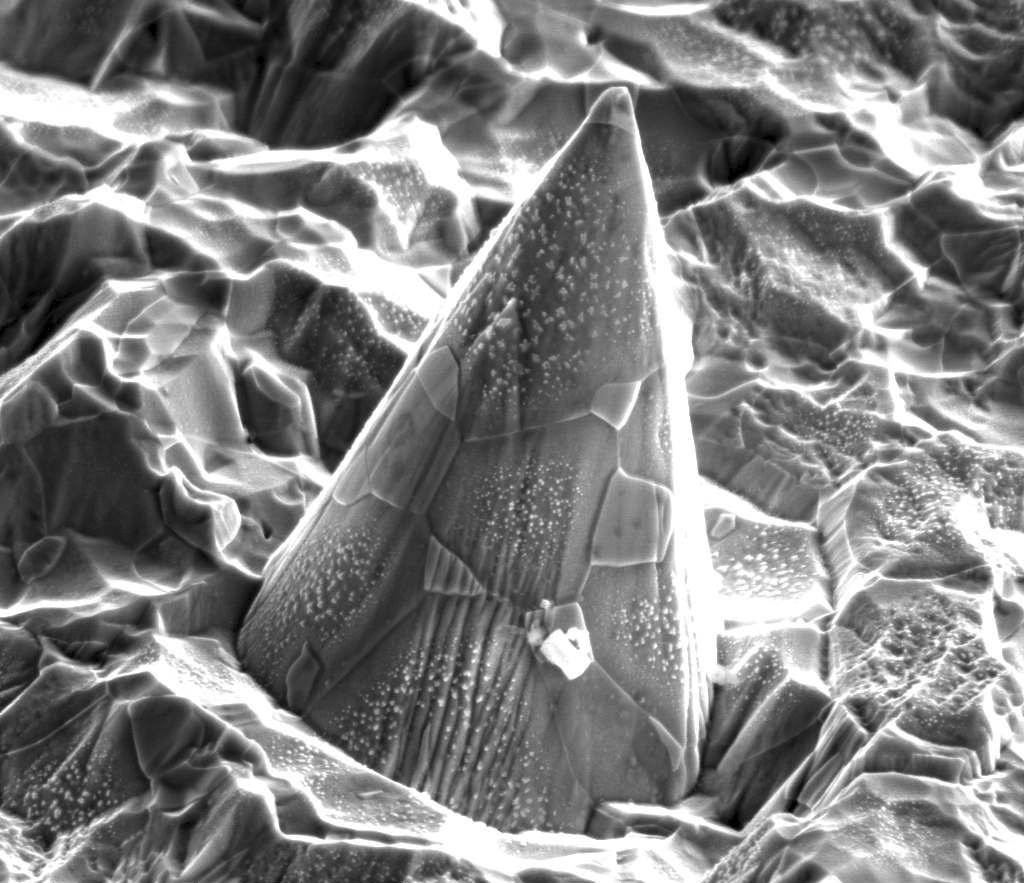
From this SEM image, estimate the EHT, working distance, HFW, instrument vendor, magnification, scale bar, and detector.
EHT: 10 kV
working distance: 10.1 mm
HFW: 24.9 µm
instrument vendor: FEI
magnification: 6 K X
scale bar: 5000 nm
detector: SE